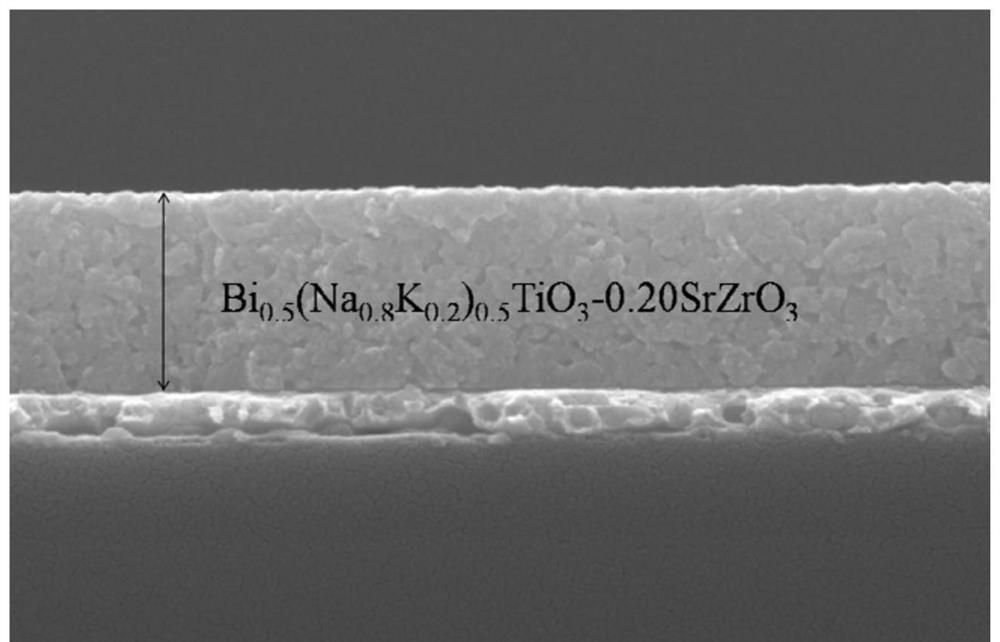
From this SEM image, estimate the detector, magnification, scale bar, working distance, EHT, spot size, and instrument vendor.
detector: SE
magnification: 40 K X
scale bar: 500 nm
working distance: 6 mm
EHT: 20 kV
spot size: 3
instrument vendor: FEI